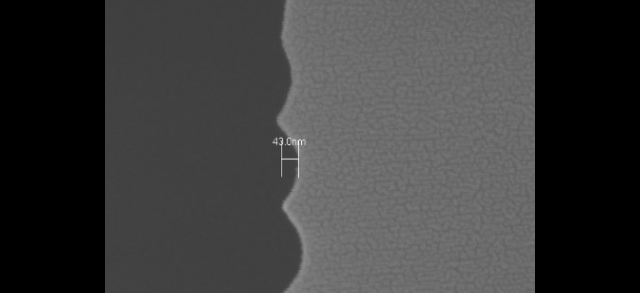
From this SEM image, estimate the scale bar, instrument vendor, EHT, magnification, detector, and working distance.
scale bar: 400 nm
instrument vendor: Hitachi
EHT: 10 kV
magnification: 120 K X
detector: SE(U)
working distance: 10.1 mm